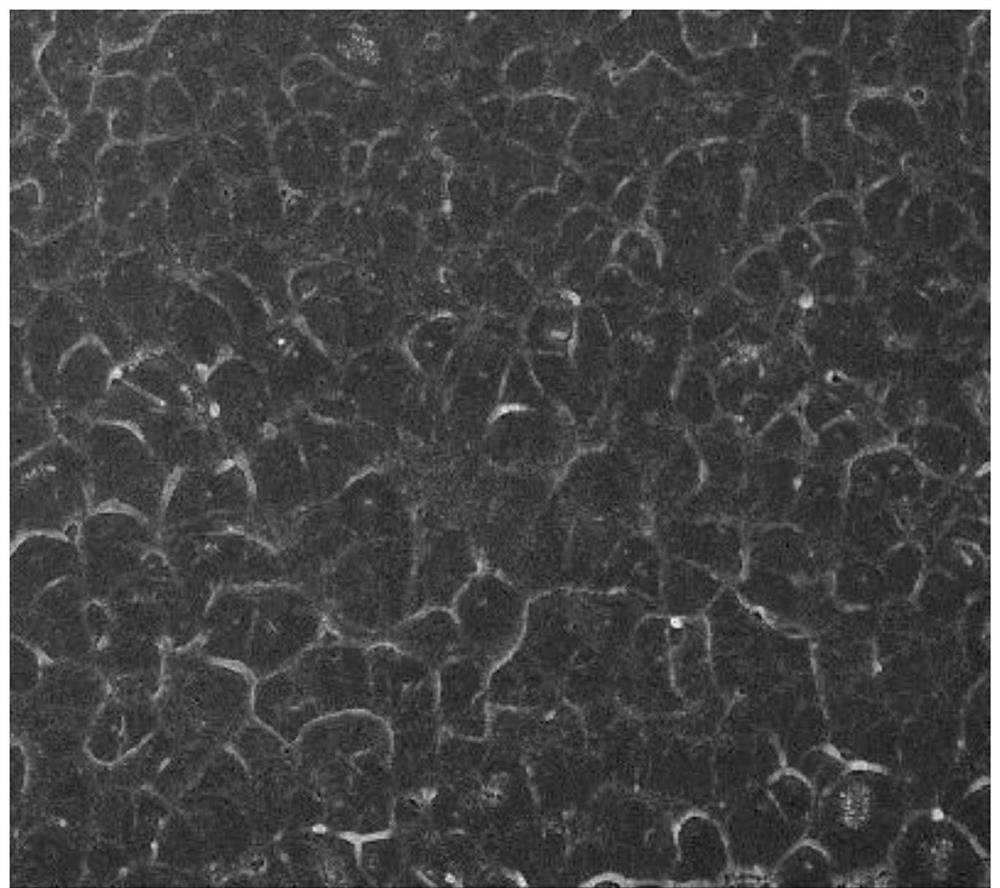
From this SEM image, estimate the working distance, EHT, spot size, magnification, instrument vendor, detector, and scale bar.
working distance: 5.6 mm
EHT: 10 kV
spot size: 4.5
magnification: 10 K X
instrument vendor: FEI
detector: TLD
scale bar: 5000 nm